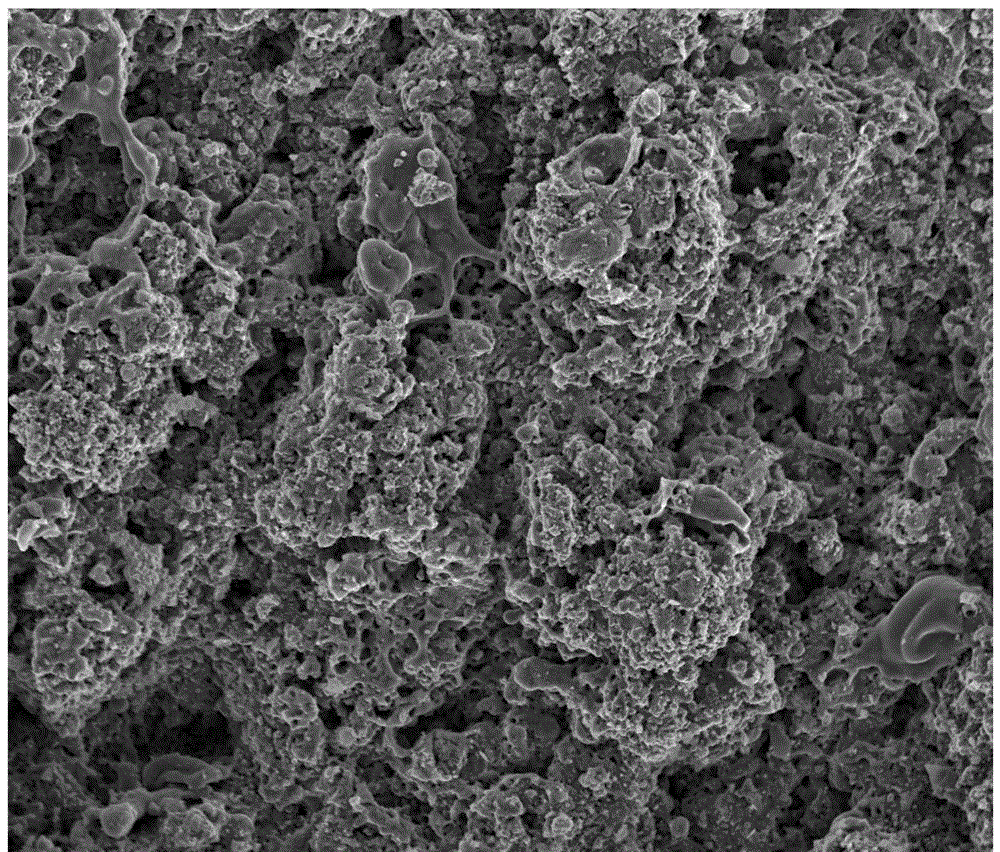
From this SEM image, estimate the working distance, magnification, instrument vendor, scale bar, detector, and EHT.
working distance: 3.5 mm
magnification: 1.5 K X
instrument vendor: FEI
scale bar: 50000 nm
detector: ETD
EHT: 10 kV